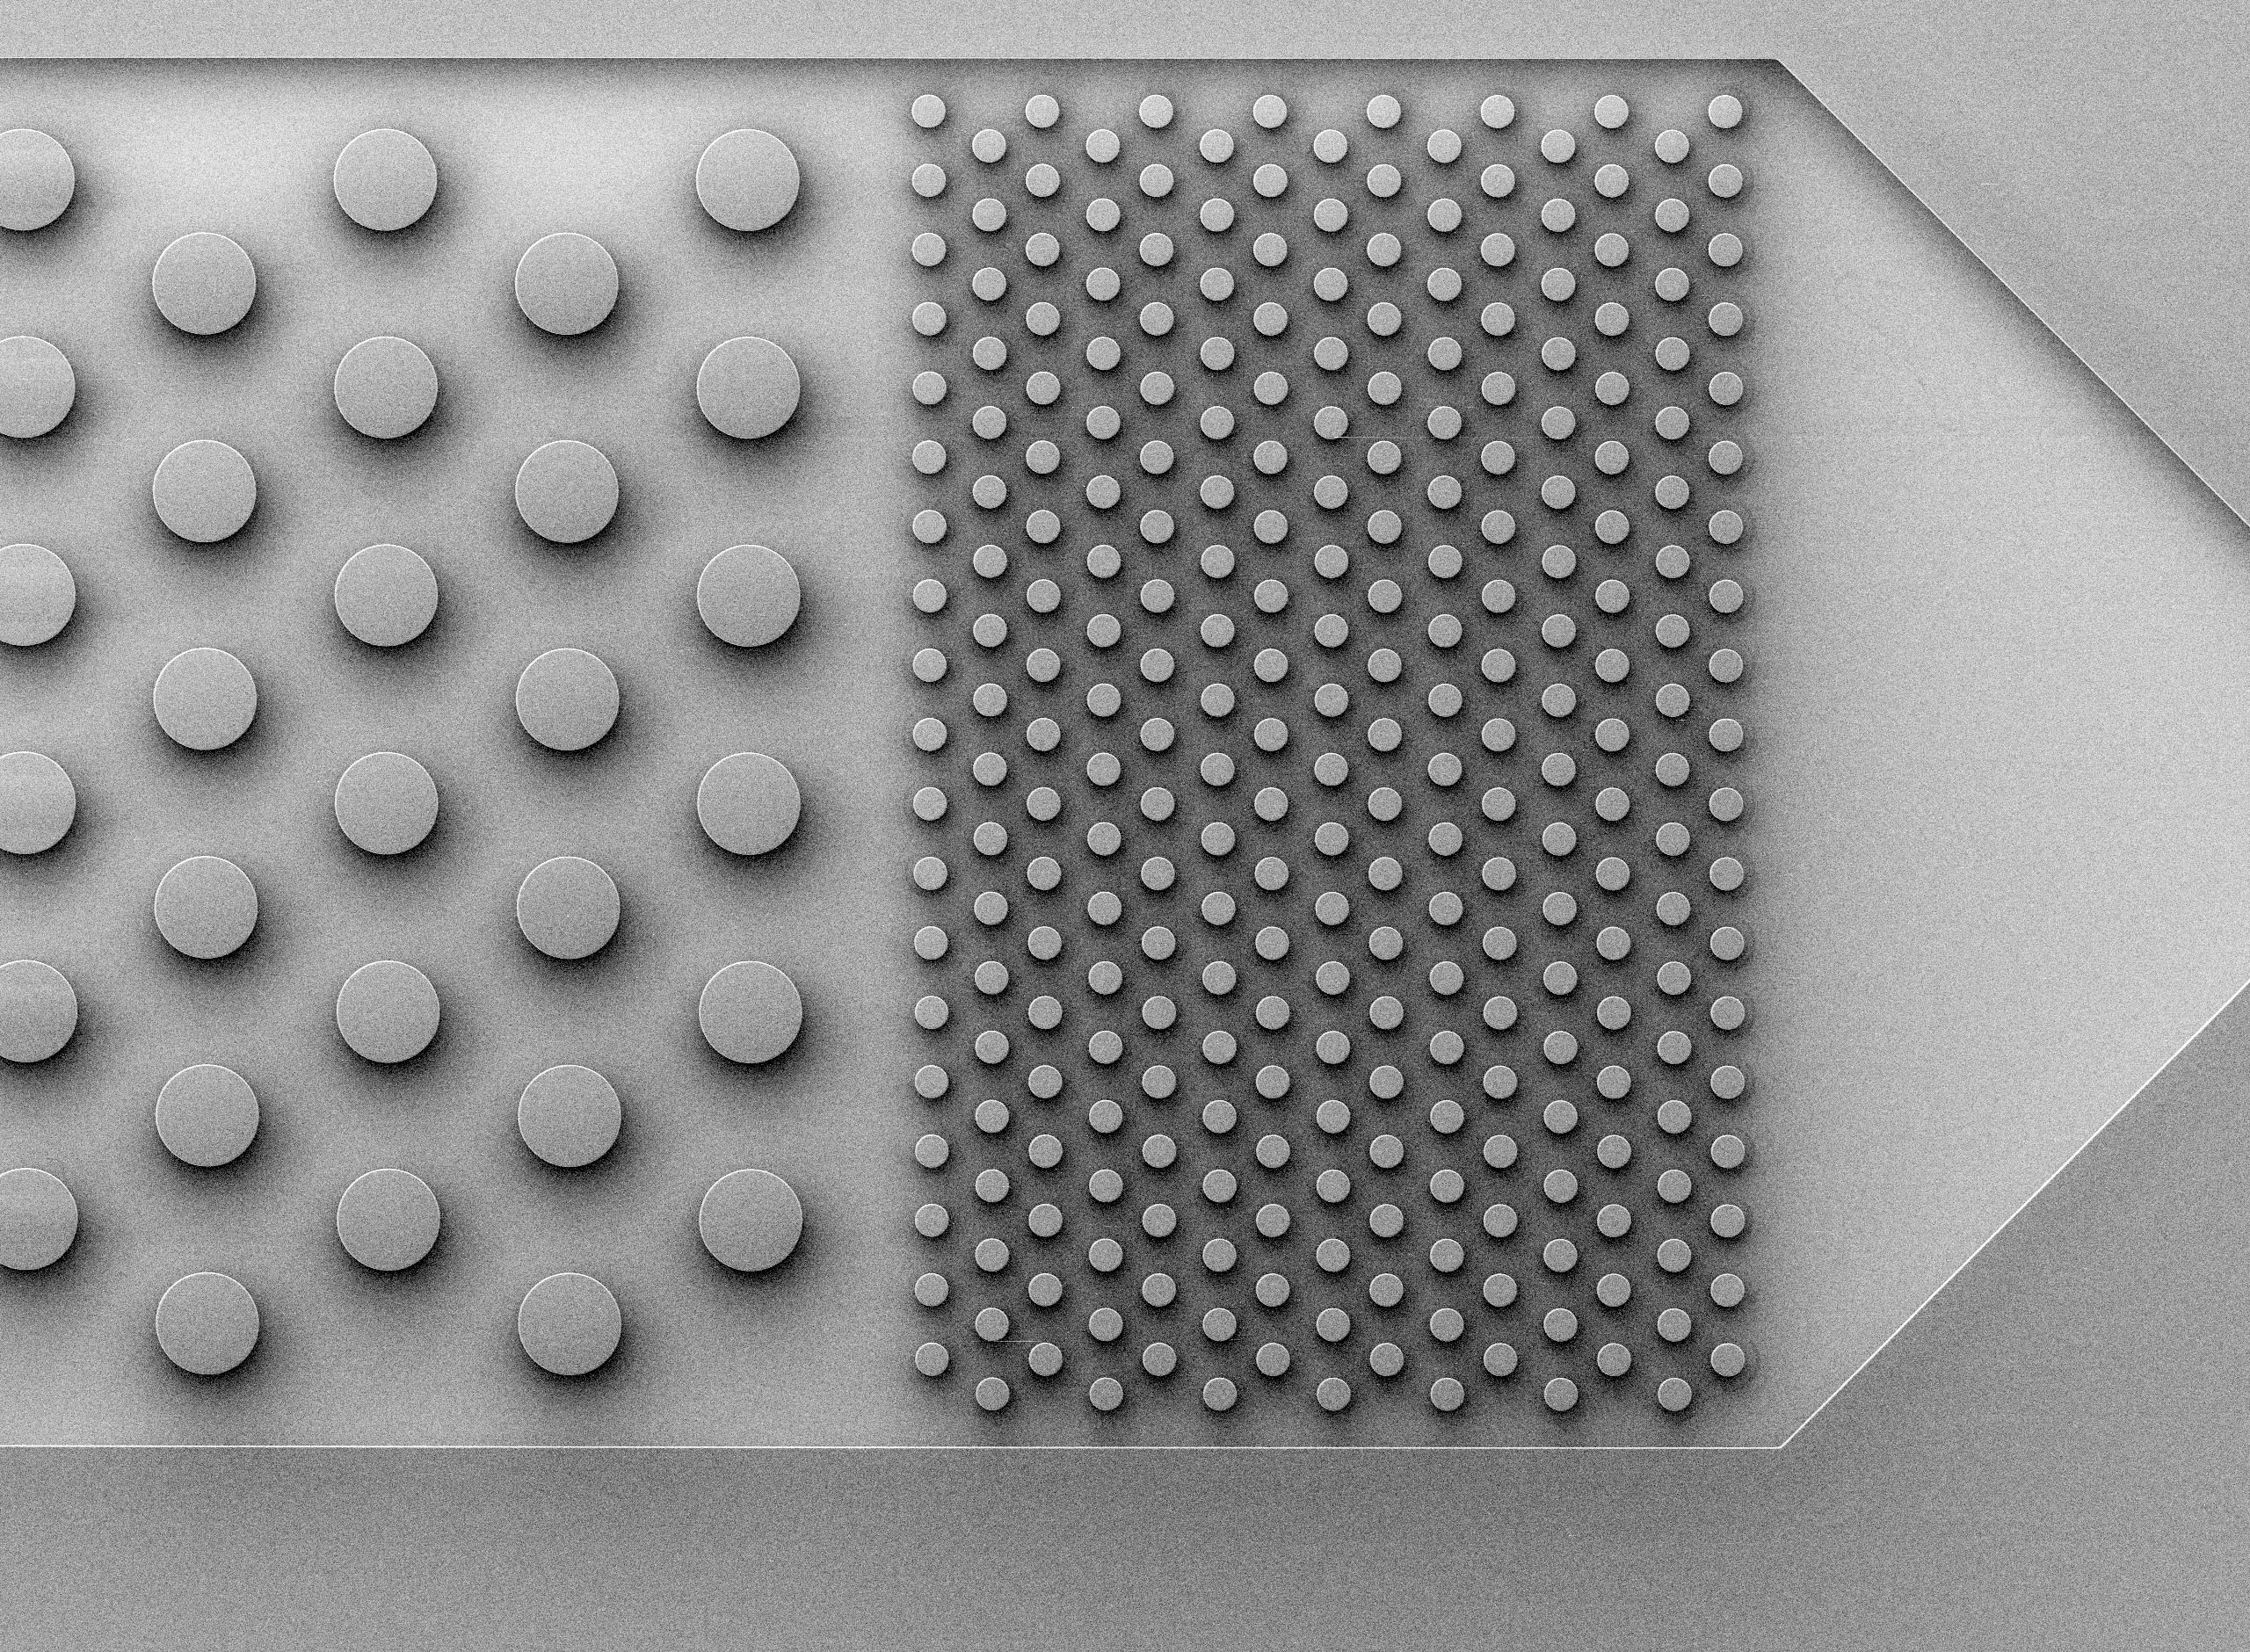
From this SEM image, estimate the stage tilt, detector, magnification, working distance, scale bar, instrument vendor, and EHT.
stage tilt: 0°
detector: SEI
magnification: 0.037 K X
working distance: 20 mm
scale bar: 100000 nm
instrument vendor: JEOL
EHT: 2 kV